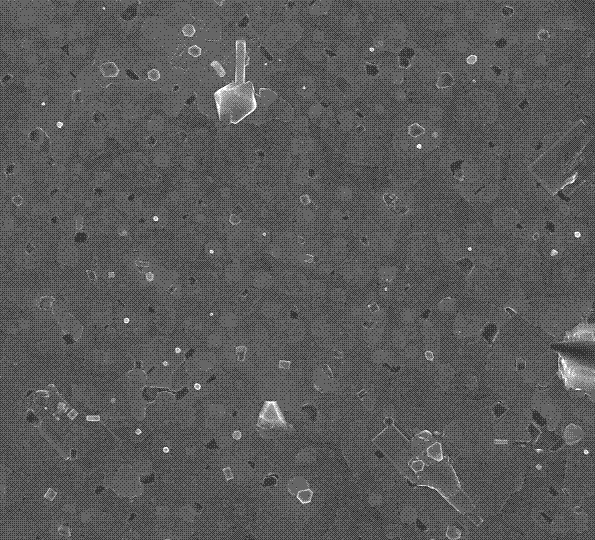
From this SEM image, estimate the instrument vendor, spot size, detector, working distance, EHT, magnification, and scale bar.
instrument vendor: FEI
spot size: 3.5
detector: TLD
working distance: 6.4 mm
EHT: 18 kV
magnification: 20 K X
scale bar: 3000 nm